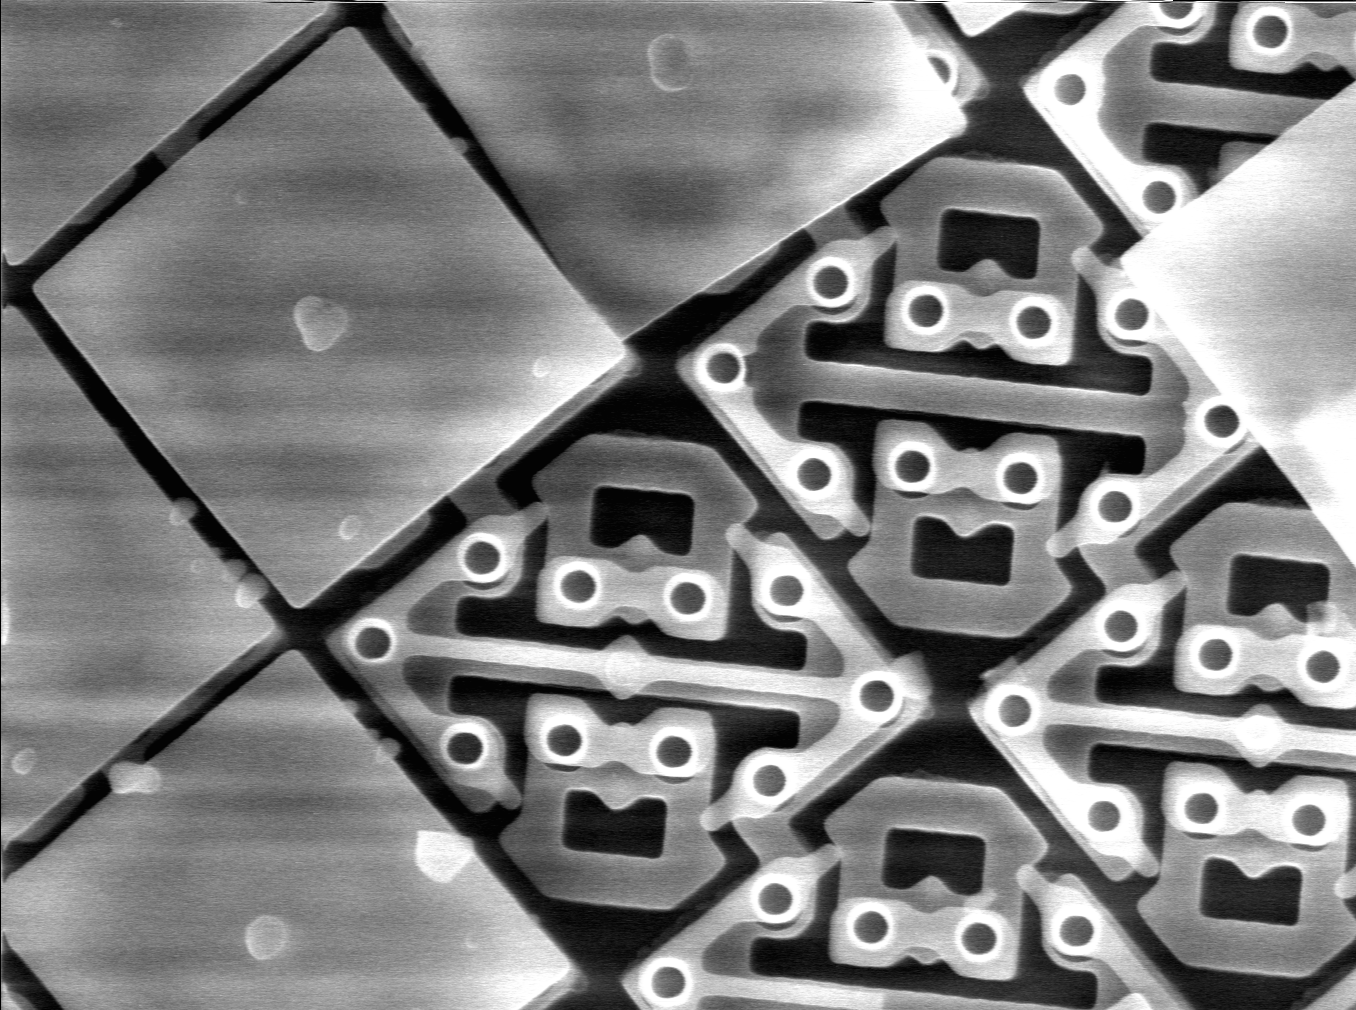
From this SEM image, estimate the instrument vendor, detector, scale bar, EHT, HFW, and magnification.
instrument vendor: other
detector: SE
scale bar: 3330 nm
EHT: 15 kV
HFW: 40 µm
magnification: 3 K X